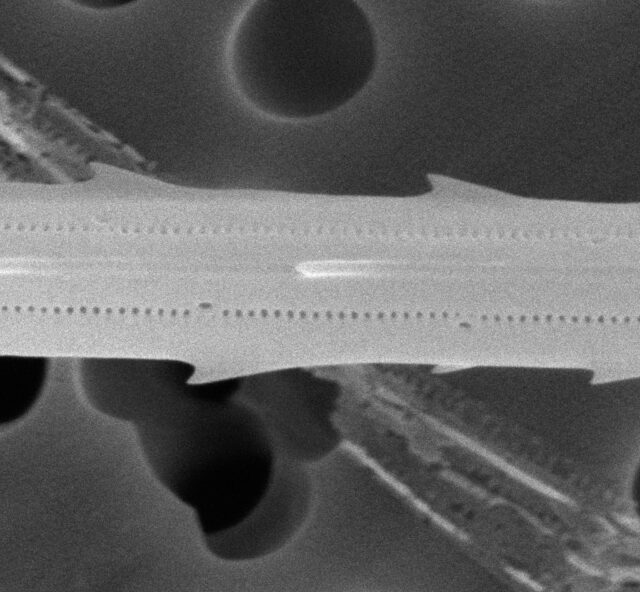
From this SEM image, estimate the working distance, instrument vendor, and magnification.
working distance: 10.7 mm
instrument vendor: JEOL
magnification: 27 K X